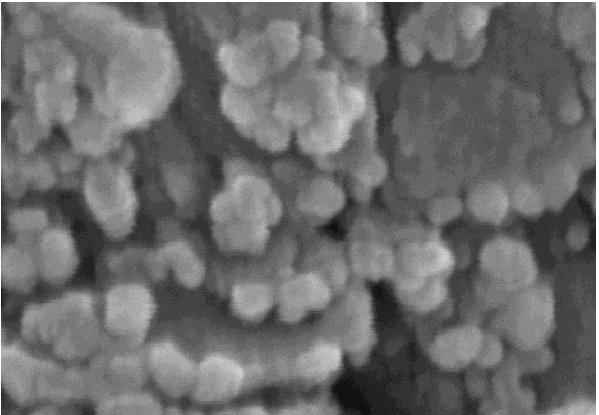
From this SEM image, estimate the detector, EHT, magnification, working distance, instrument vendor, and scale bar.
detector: SE(U)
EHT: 10 kV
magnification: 400 K X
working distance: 9.5 mm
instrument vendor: Hitachi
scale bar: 100 nm